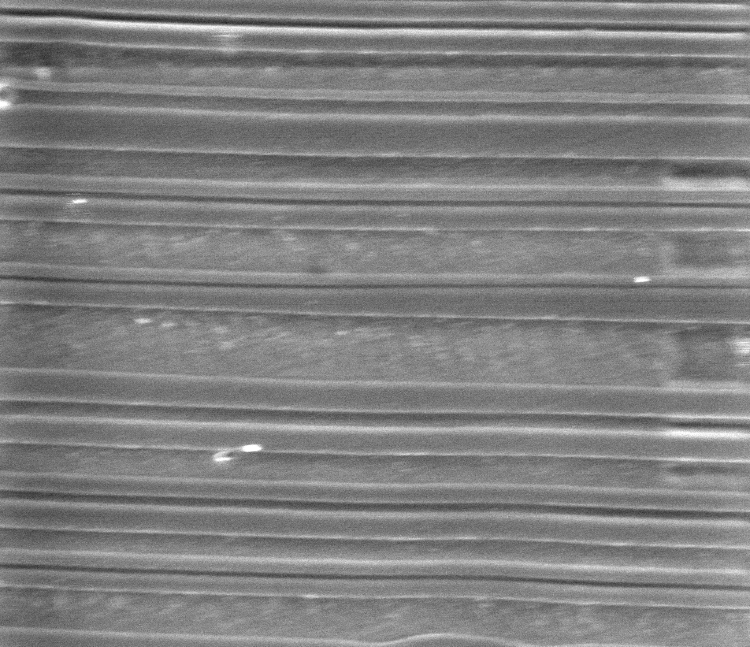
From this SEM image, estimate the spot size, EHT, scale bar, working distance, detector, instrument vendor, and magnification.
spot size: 3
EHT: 5 kV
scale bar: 10000 nm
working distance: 11.1 mm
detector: SE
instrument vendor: FEI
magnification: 9.154 K X